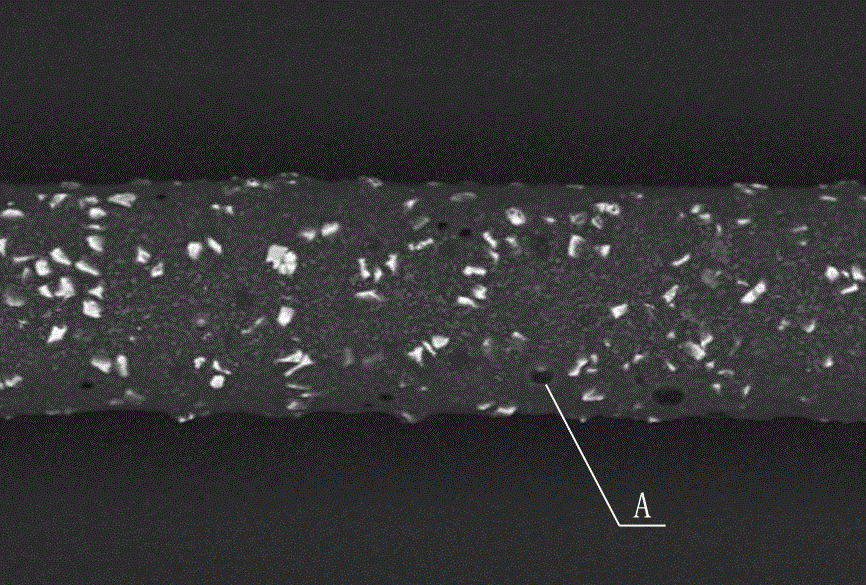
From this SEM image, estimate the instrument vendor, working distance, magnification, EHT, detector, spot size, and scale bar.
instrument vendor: JEOL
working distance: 10 mm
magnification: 0.3 K X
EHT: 15 kV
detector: BEC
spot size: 50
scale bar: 50000 nm